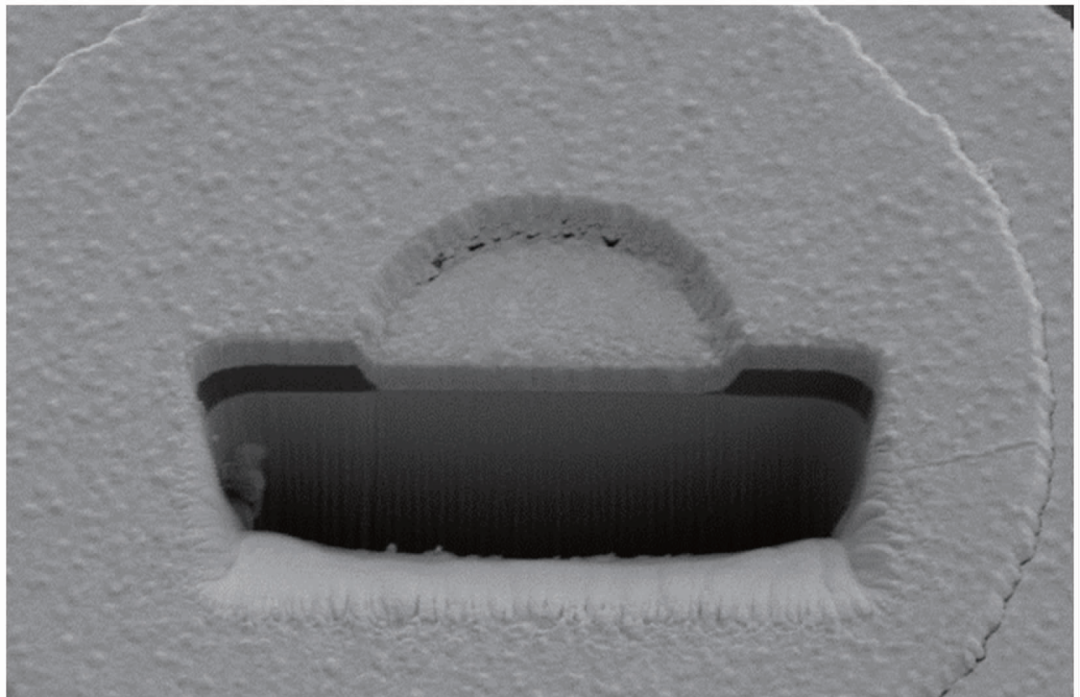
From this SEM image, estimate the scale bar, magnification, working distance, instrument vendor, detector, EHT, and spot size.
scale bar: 5000 nm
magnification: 6.405 K X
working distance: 4.9 mm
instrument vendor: FEI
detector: SE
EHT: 5 kV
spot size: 3